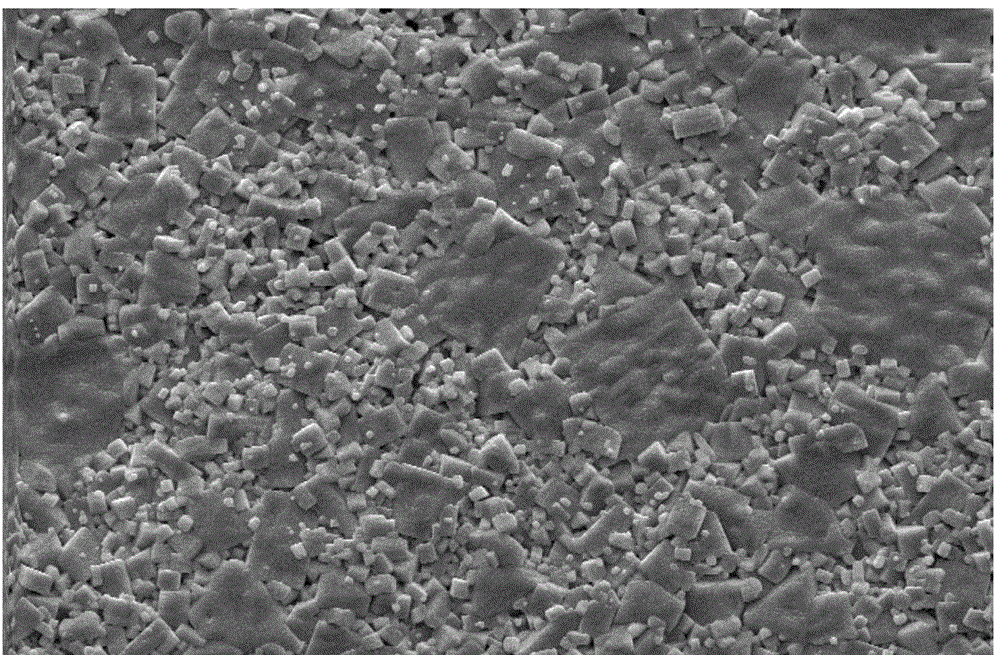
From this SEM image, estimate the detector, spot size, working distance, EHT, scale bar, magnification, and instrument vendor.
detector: SE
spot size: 3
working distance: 6.2 mm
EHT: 20 kV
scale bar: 20000 nm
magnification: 1 K X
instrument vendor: FEI